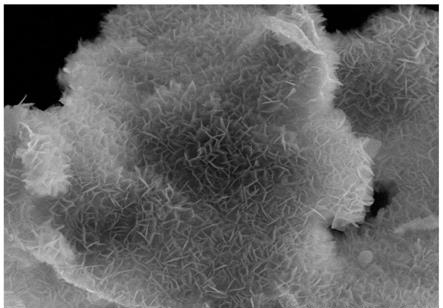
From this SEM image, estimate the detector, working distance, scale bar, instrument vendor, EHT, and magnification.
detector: SE(M)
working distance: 10.4 mm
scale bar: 2000 nm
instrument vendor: Hitachi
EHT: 5 kV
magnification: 20 K X